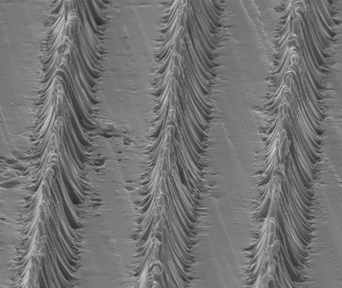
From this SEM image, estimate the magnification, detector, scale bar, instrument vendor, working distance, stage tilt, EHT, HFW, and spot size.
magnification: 5 K X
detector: ETD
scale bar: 10000 nm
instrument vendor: FEI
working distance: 14.5 mm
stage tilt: -15°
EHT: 10 kV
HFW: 59.7 µm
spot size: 3